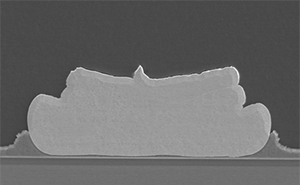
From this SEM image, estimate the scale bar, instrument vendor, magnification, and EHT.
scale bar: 10000 nm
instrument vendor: JEOL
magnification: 1.5 K X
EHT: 10 kV